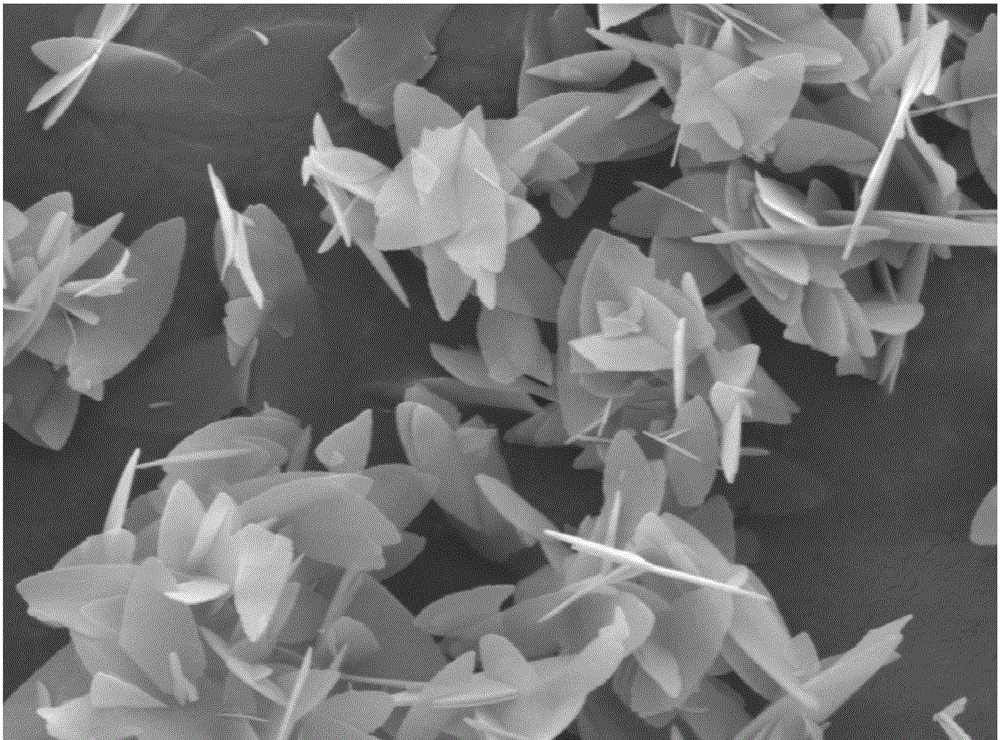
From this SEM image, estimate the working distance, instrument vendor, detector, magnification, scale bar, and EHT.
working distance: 10.1 mm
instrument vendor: JEOL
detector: LED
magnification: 5 K X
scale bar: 1000 nm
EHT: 8 kV